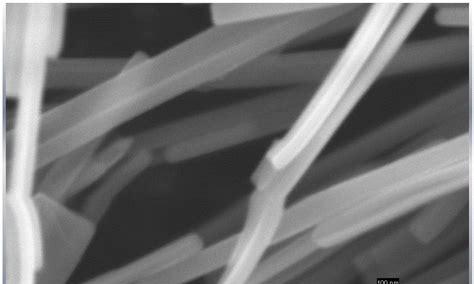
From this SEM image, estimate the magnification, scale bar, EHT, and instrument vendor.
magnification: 40.45 K X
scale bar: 100 nm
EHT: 10 kV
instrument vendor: Zeiss